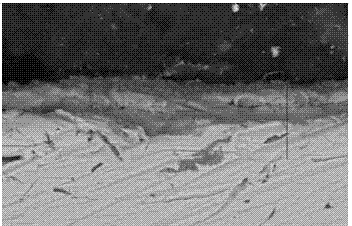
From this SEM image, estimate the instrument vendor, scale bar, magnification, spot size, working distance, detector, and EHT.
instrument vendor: FEI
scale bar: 10000 nm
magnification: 2 K X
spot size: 4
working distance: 7.5 mm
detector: BSE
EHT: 20 kV